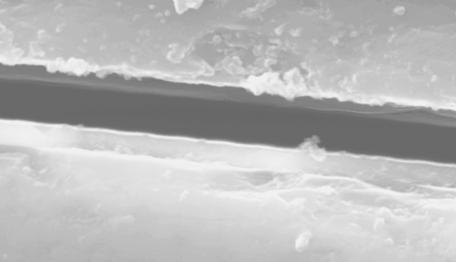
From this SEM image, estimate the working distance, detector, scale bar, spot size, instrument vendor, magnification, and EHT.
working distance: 9.6 mm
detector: SE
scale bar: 2000 nm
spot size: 4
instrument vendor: FEI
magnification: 10 K X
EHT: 20 kV